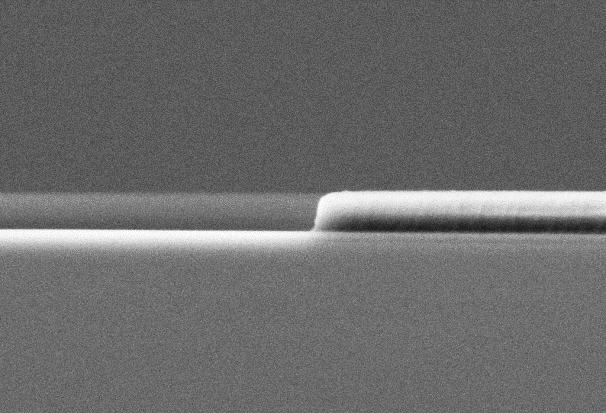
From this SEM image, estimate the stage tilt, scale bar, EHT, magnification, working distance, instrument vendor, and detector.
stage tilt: -1°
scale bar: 200 nm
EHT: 5 kV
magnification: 50 K X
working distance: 4.7 mm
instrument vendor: Zeiss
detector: SE2